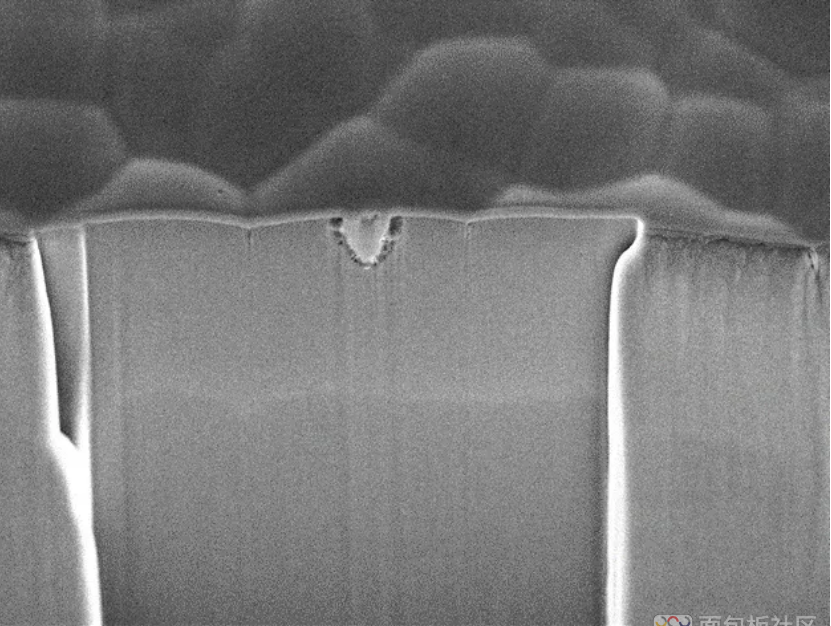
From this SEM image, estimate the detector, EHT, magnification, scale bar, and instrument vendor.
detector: SEI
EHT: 10 kV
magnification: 7 K X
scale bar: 1000 nm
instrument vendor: JEOL